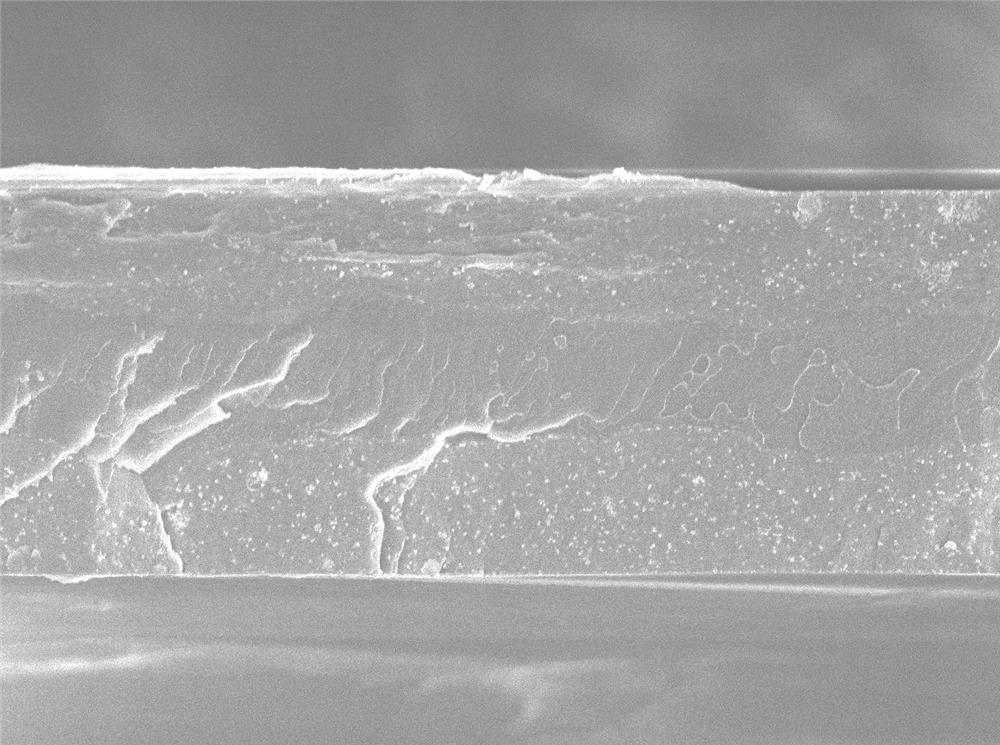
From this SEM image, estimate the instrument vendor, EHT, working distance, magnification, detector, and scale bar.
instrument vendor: JEOL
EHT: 5 kV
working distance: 7.8 mm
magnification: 3 K X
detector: SEI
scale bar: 1000 nm